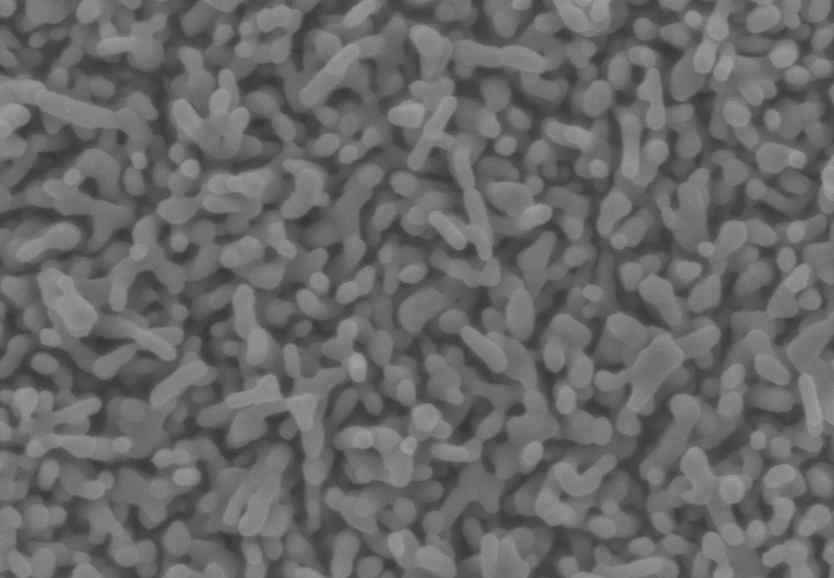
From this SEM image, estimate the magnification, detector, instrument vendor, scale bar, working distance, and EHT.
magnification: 30 K X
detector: SE(U)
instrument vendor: Hitachi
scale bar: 1000 nm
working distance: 10 mm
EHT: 5 kV